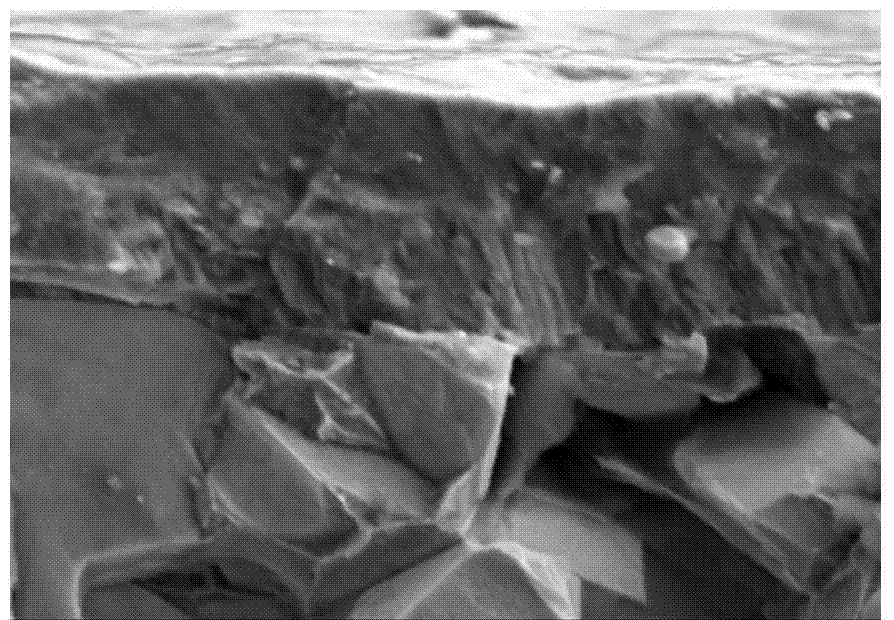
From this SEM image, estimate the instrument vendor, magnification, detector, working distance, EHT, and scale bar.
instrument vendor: Hitachi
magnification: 20 K X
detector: SE(M,LA0)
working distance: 9.6 mm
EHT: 5 kV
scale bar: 2000 nm